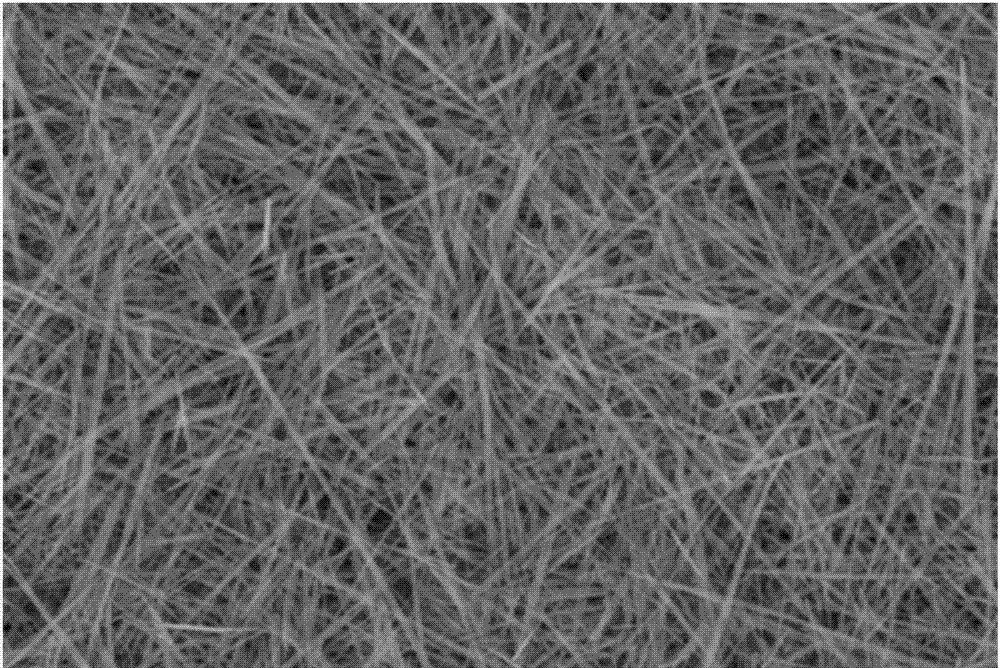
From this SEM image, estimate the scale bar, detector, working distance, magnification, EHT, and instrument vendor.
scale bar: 1000 nm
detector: SE2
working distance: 8.8 mm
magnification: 20 K X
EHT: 5 kV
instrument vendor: Zeiss